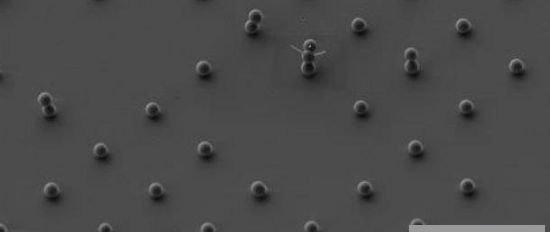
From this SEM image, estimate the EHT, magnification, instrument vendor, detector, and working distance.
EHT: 3 kV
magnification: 3 K X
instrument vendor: Zeiss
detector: SE2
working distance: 4.4 mm